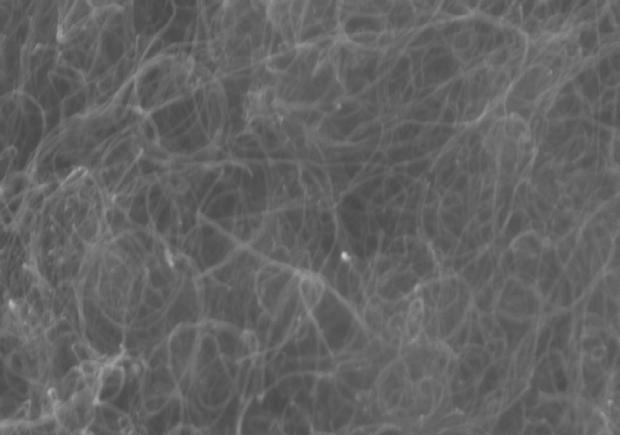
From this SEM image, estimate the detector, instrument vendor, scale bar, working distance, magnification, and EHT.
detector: InLens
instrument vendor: Zeiss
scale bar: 200 nm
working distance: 5 mm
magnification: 40 K X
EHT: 15 kV